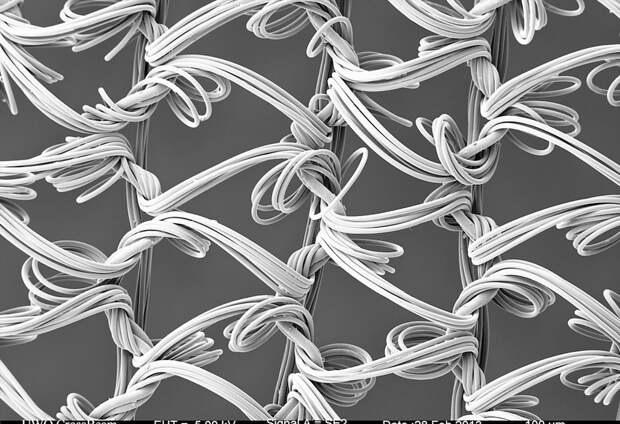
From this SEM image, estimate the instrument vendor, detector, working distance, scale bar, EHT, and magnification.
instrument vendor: Zeiss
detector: SE2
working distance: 19.4 mm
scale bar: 100000 nm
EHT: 5 kV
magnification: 0.05 K X